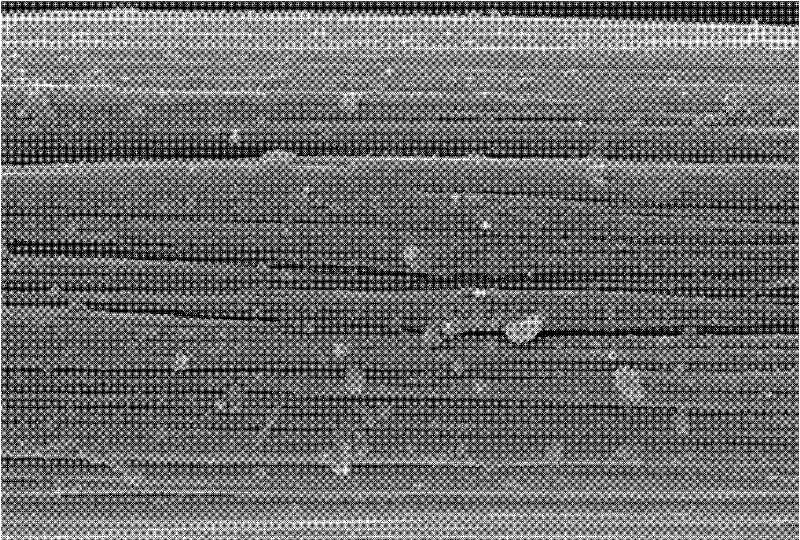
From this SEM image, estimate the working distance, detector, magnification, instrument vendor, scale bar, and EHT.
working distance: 5.4 mm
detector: SE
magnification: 10 K X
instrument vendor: Hitachi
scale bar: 5000 nm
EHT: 15 kV